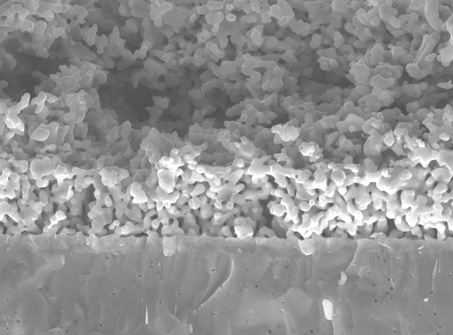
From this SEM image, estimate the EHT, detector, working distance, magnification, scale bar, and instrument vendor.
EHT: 20 kV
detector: SEI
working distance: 12 mm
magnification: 2.5 K X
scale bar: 10000 nm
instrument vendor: JEOL